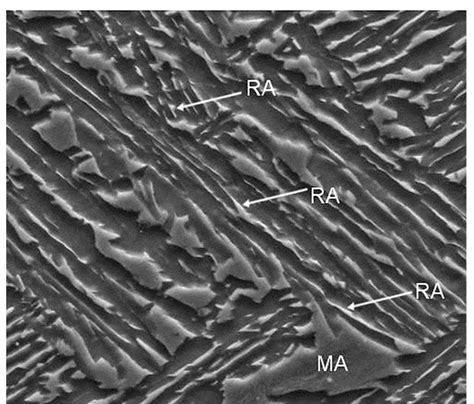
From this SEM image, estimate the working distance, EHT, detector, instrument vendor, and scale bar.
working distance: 9.9 mm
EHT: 15 kV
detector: ETD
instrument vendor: FEI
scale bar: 10000 nm